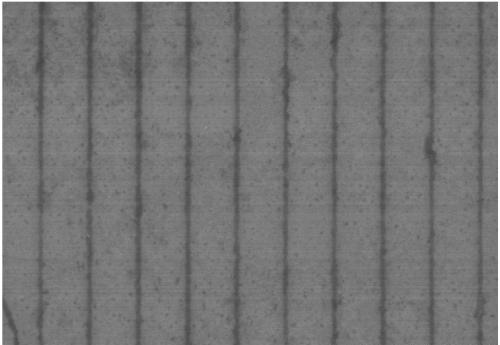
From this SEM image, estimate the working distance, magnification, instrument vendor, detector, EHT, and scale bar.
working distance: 9.1 mm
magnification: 20 K X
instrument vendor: Hitachi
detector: SE(M)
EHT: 3 kV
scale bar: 2000 nm